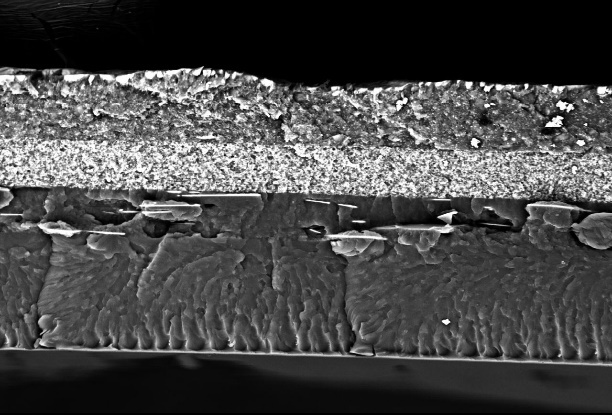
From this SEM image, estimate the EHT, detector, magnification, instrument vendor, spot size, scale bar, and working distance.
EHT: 15 kV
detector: BSE-COMPO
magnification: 1 K X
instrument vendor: other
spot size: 16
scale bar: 10000 nm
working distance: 13.7 mm